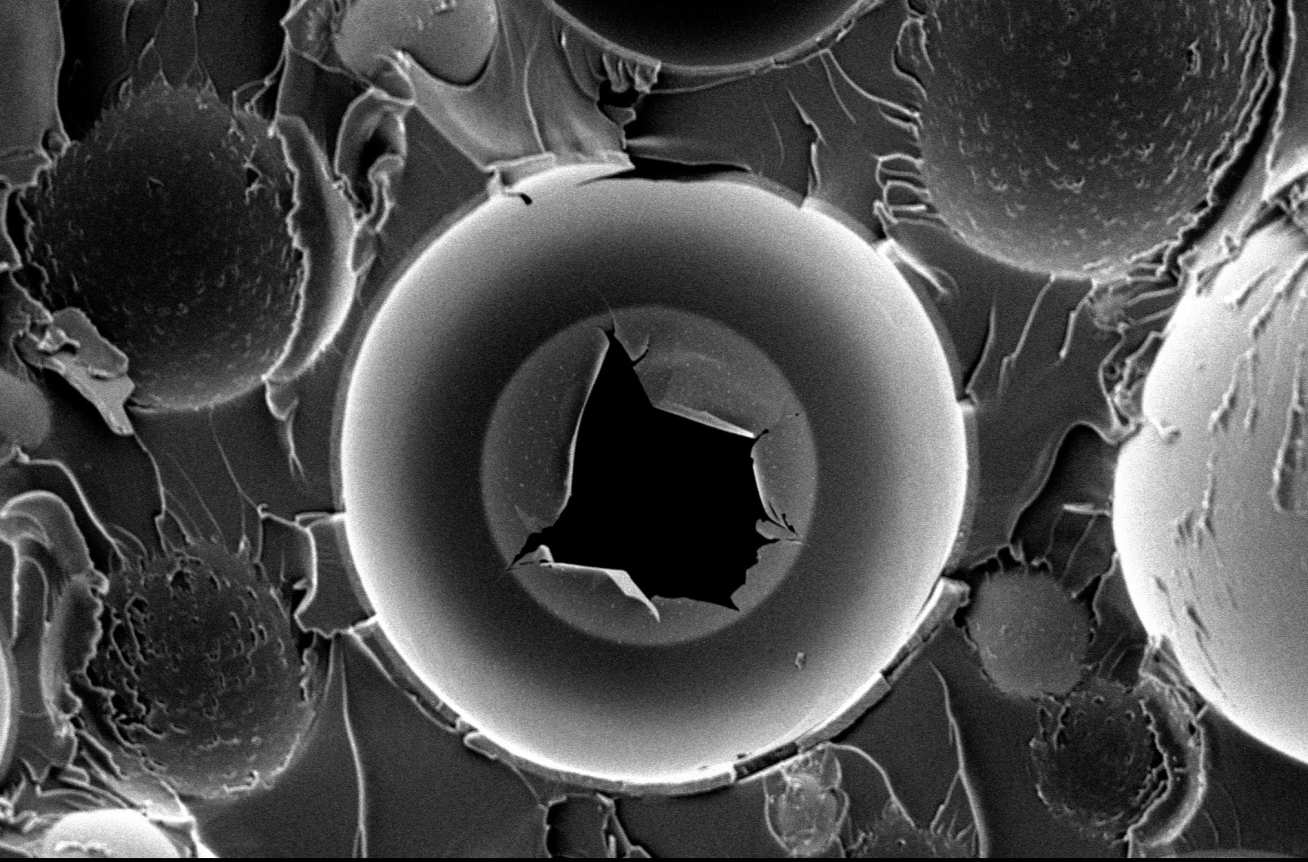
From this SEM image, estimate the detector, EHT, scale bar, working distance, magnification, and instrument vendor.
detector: SE2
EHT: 3 kV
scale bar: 10000 nm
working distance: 9.6 mm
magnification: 3 K X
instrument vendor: Zeiss